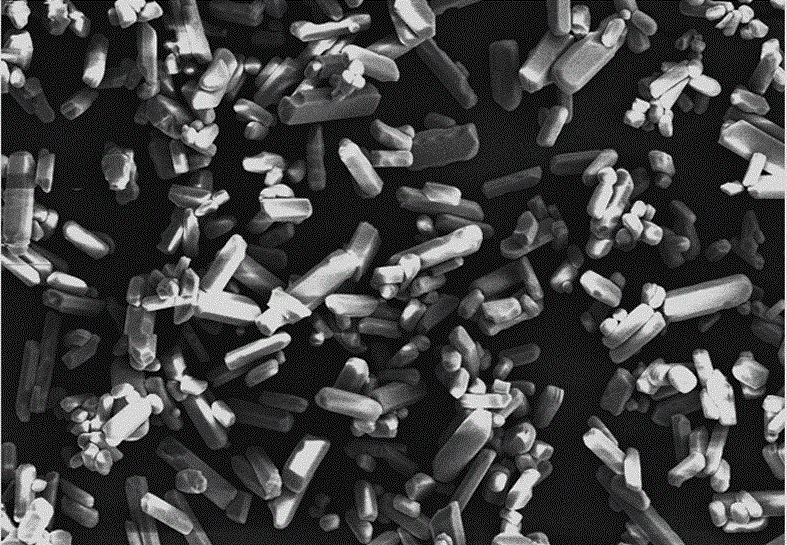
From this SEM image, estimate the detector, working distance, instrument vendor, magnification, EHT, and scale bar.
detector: SE(M)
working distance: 11.7 mm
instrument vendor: Hitachi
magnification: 0.5 K X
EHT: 15 kV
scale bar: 50000 nm